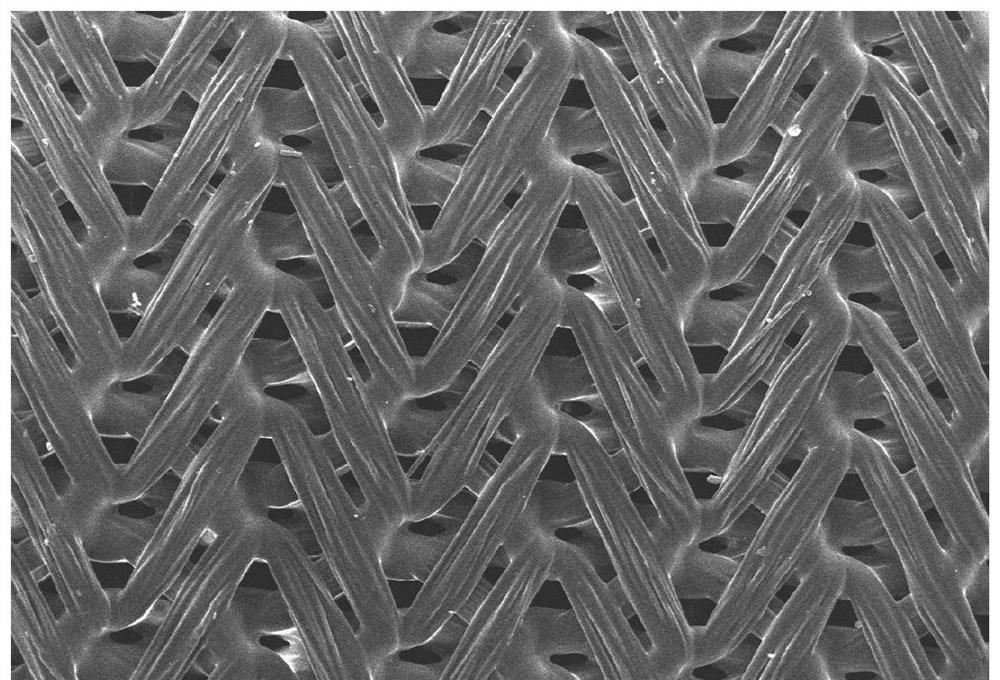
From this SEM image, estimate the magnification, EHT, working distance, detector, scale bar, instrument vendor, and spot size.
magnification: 0.04 K X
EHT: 30 kV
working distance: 11 mm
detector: SEI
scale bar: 500000 nm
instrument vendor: JEOL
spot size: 30